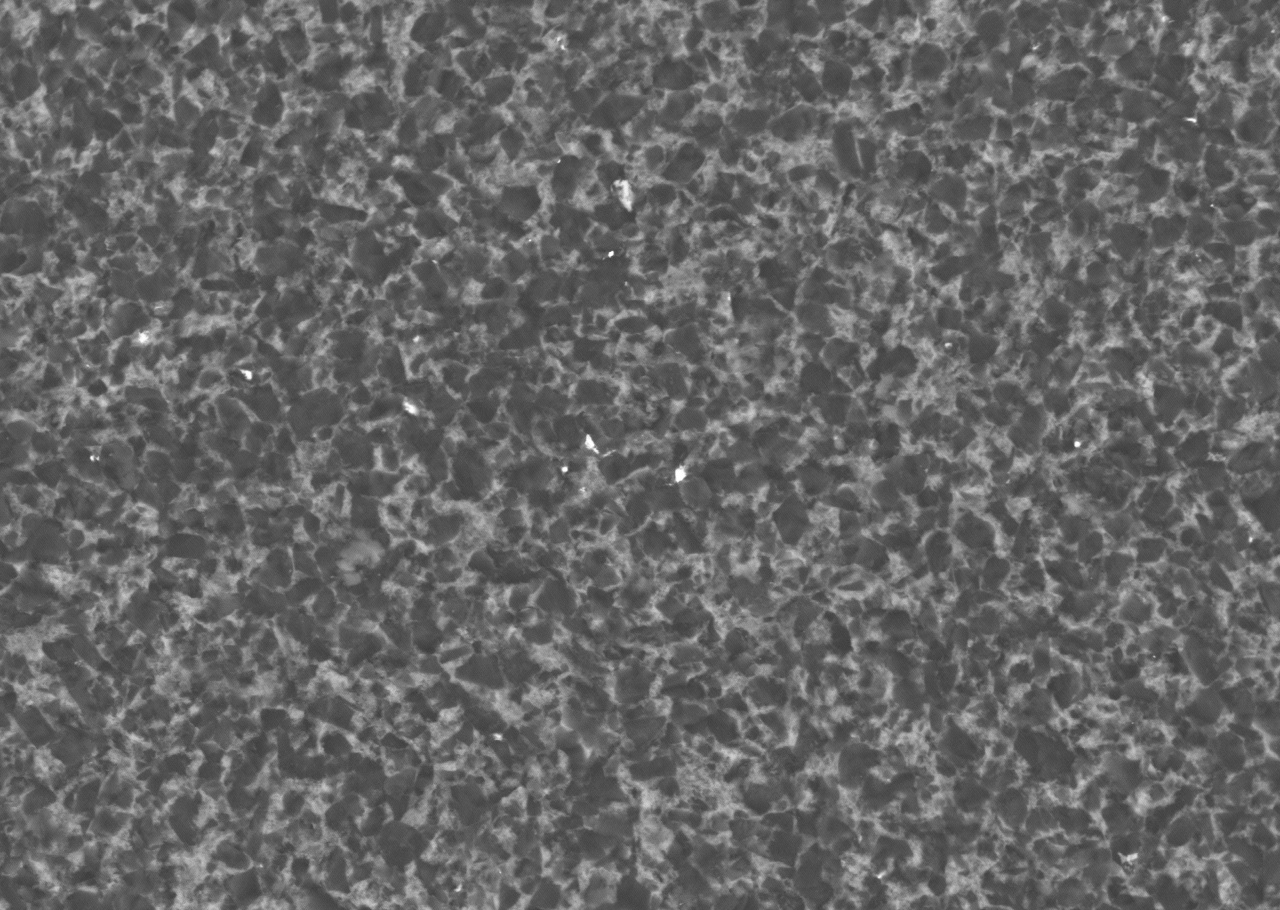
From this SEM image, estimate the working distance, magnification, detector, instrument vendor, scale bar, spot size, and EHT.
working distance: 7.9 mm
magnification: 1.5 K X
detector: BSEI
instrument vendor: other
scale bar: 10000 nm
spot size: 13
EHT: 20 kV